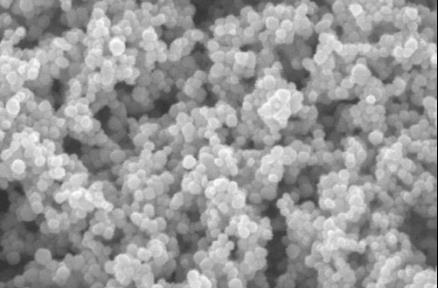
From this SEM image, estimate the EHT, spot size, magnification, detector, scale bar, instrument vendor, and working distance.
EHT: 5 kV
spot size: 4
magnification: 200 K X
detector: TLD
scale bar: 200 nm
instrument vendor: FEI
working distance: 6.6 mm